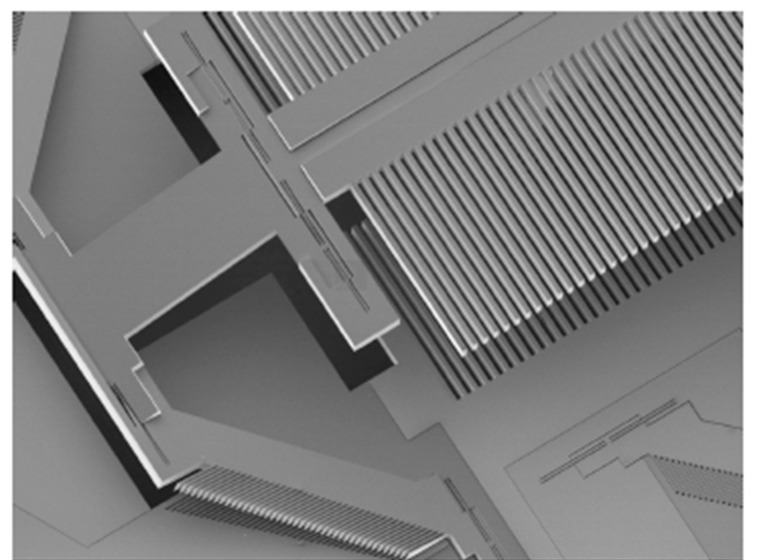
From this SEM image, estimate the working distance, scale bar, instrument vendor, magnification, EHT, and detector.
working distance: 15 mm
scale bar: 100000 nm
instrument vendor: JEOL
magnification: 0.03 K X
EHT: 5 kV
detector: LEI